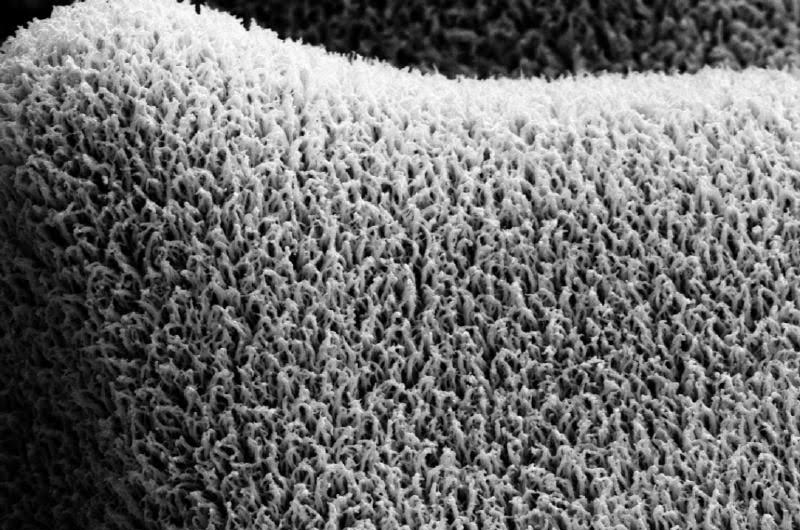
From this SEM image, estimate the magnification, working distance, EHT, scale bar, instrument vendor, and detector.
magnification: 5 K X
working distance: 15.5 mm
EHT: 20 kV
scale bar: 1000 nm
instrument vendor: Zeiss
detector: SE2